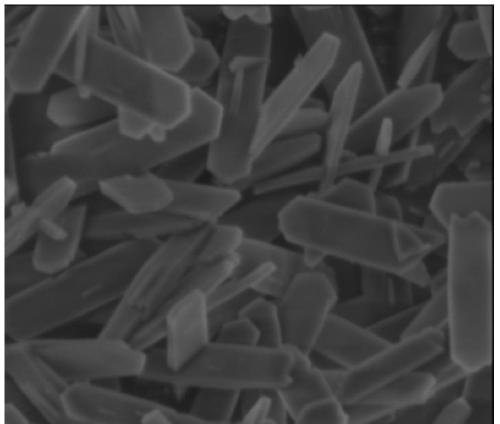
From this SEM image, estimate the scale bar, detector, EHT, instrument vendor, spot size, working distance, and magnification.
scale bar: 2000 nm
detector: ETD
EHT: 3 kV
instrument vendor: FEI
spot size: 3.5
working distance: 4.6 mm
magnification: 40 K X